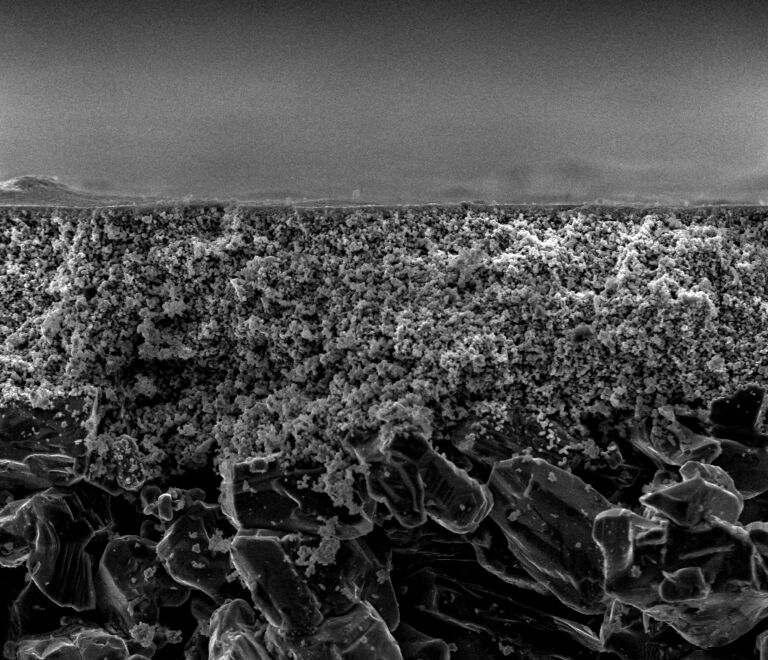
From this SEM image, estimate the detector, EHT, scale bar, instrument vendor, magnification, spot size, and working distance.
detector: ETD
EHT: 15 kV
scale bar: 100000 nm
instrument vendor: FEI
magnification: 1.037 K X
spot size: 3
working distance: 9.3 mm